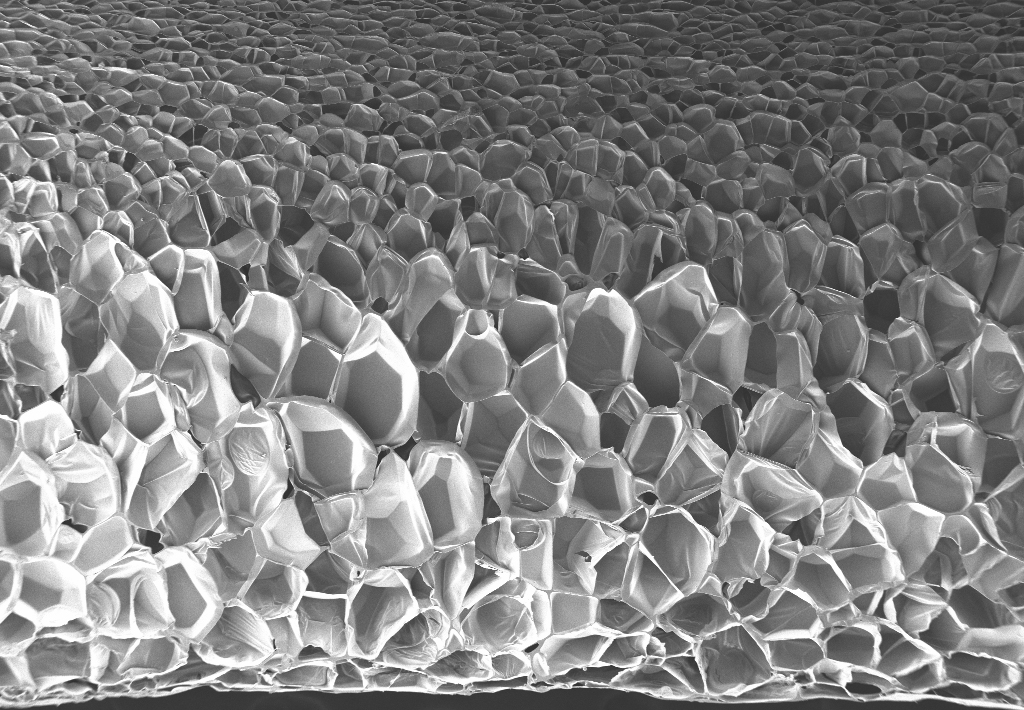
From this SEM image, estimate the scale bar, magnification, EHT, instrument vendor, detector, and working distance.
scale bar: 200000 nm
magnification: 0.05 K X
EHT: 3 kV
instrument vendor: Zeiss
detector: SE2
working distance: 13.4 mm